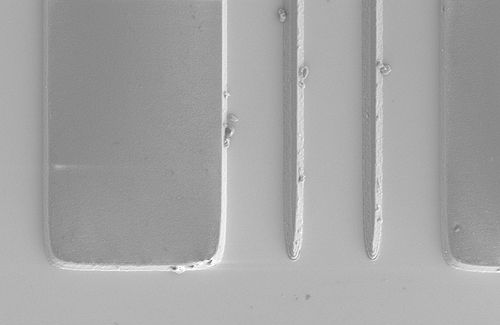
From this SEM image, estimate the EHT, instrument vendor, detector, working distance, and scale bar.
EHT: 2 kV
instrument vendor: Zeiss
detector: SE2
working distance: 8.6 mm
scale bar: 2000 nm